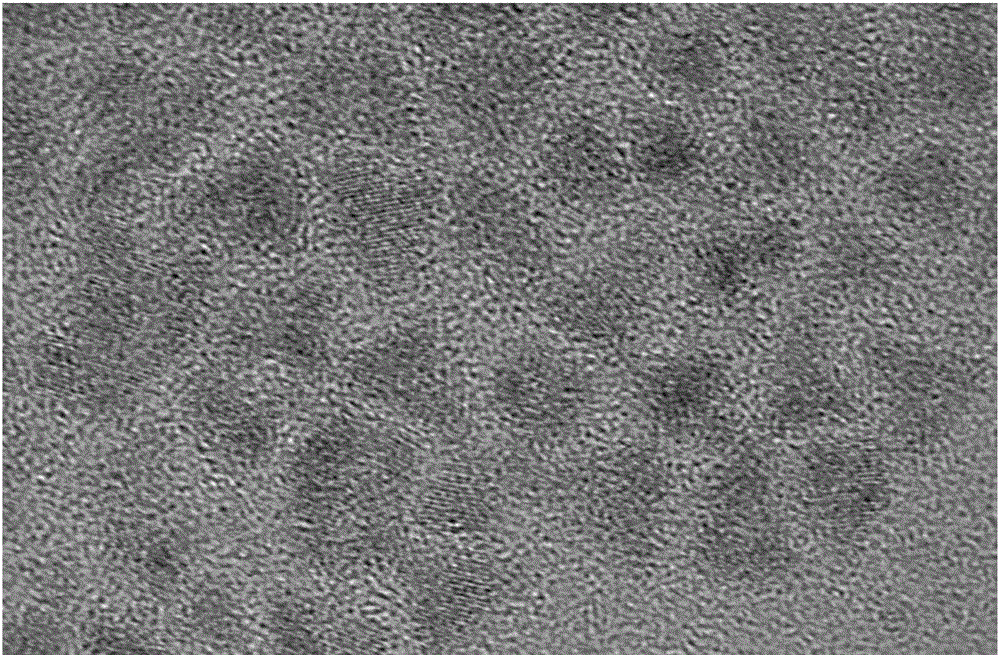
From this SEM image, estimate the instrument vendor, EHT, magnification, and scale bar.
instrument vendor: JEOL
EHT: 200 kV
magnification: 800 K X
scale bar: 10 nm